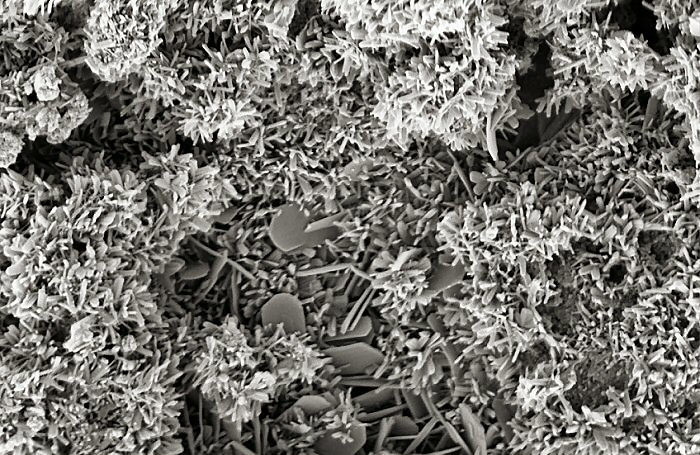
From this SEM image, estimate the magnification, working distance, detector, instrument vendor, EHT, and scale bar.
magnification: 75 K X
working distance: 4 mm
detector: InLens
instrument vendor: Zeiss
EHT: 2 kV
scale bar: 200 nm